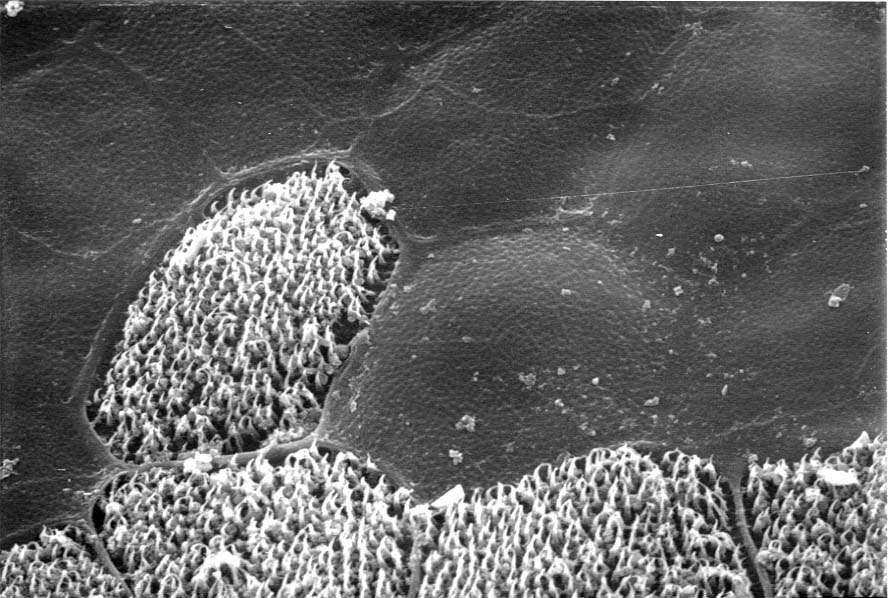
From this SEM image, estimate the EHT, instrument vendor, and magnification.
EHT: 20 kV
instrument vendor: JEOL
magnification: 2.5 K X